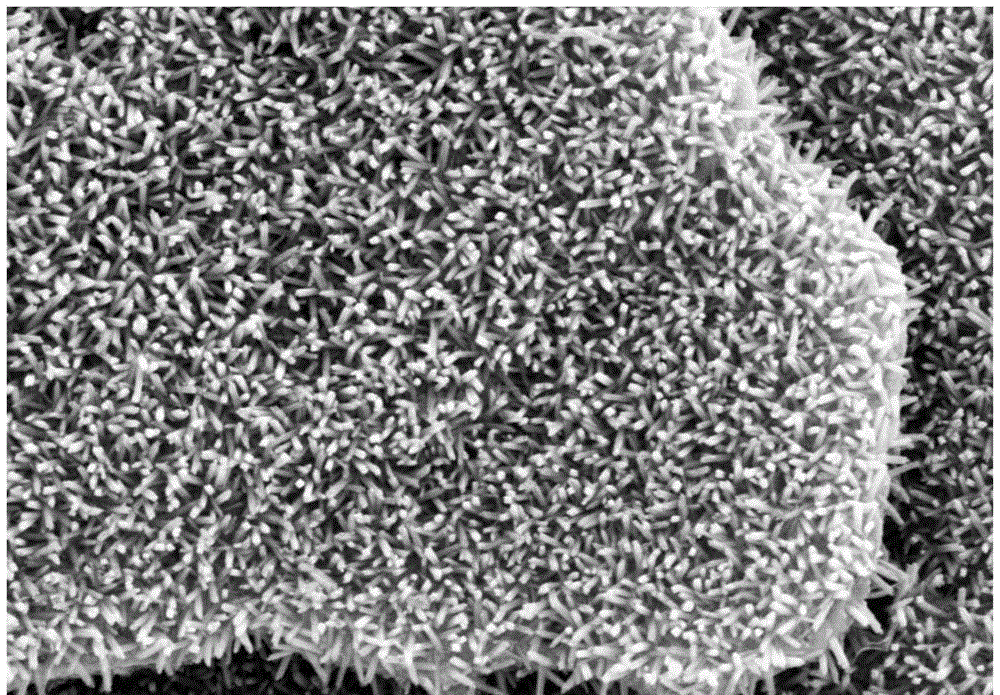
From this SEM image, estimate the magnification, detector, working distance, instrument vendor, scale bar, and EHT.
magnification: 28.8 K X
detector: SE2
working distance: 8.2 mm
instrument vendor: Zeiss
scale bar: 200 nm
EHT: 5 kV